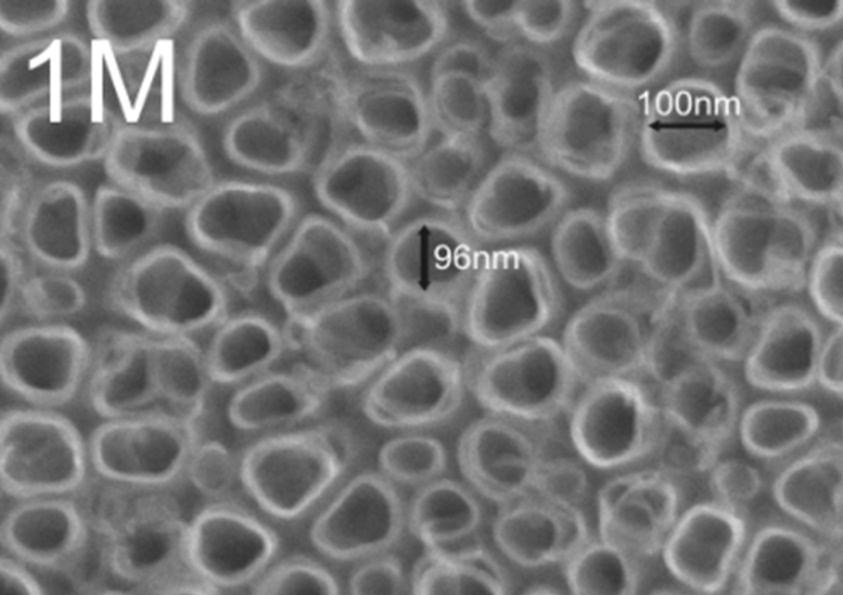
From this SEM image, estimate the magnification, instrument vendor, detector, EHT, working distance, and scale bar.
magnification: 100 K X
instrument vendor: JEOL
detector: SEI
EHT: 12 kV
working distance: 8.3 mm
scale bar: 100 nm